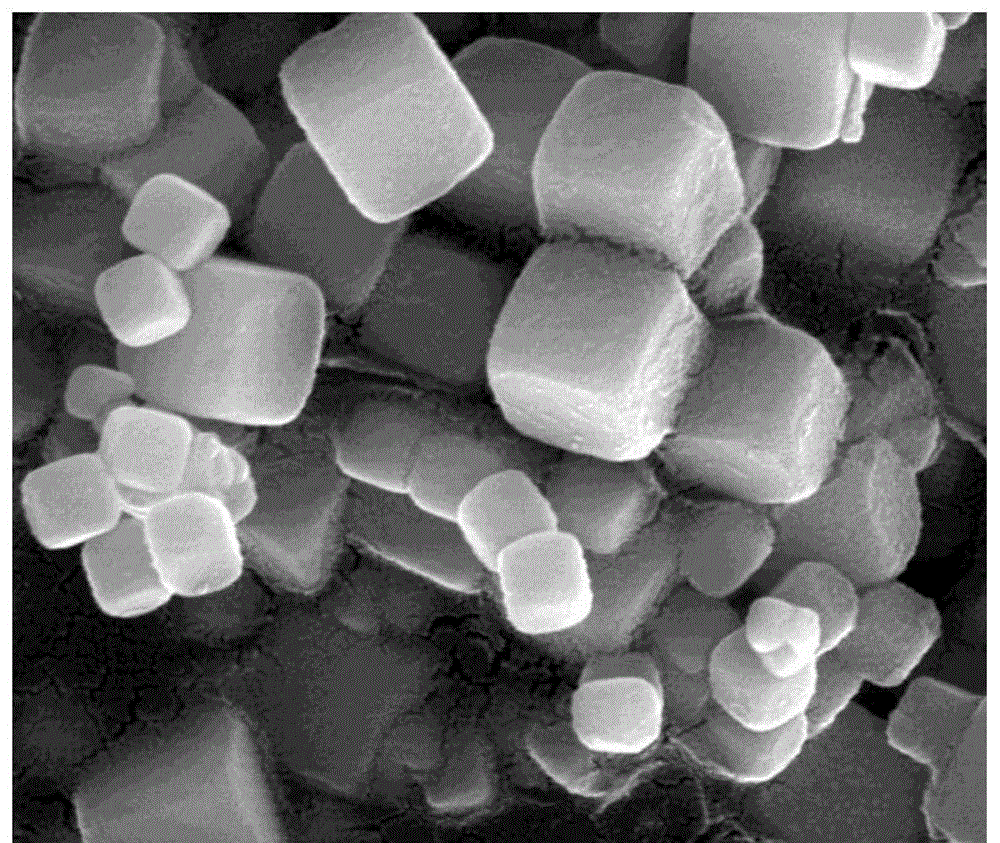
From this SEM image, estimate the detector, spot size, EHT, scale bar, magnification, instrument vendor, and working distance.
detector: ETD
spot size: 3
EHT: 20 kV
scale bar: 500 nm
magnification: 120 K X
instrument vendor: FEI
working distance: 10 mm